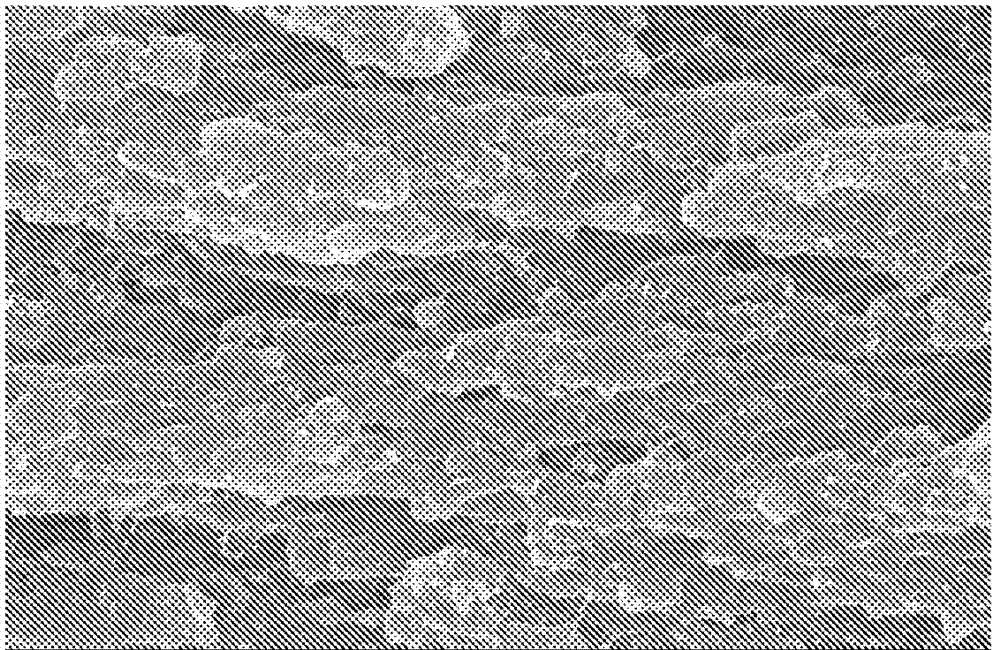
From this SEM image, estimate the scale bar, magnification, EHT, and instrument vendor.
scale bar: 2000 nm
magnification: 6 K X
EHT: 20 kV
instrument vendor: JEOL